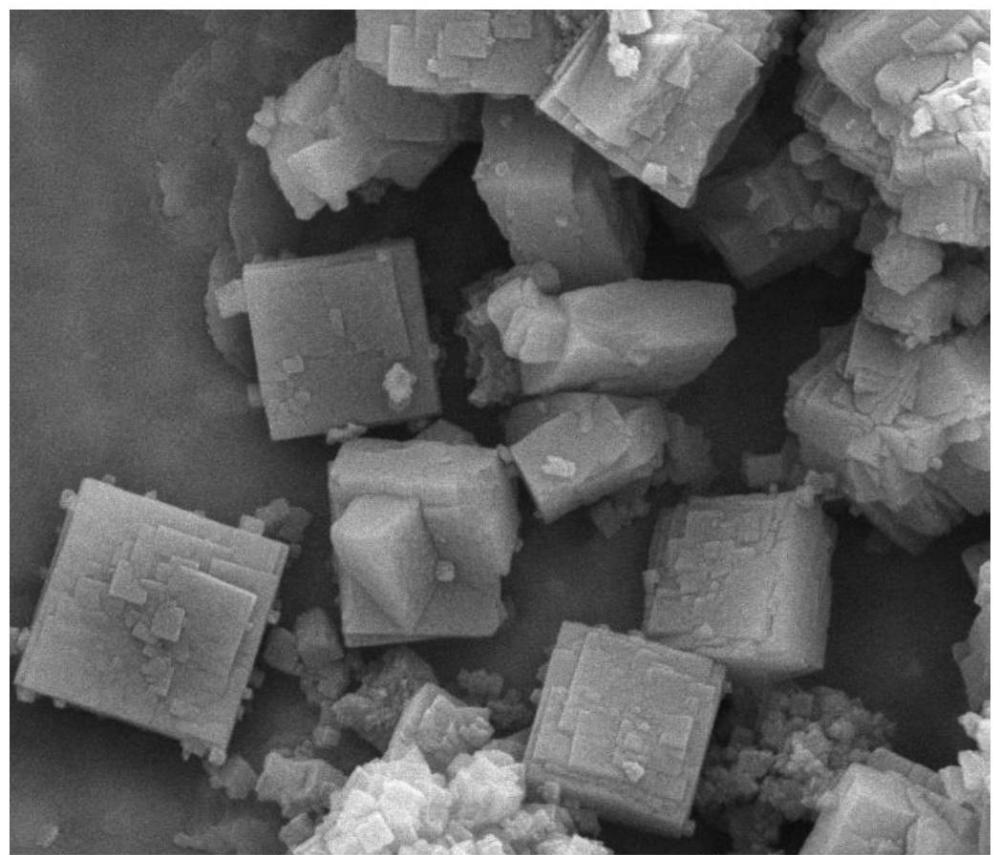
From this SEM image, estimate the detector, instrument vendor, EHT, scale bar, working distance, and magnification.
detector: ETD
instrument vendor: FEI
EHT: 10 kV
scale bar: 5000 nm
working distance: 11.1 mm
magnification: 20 K X